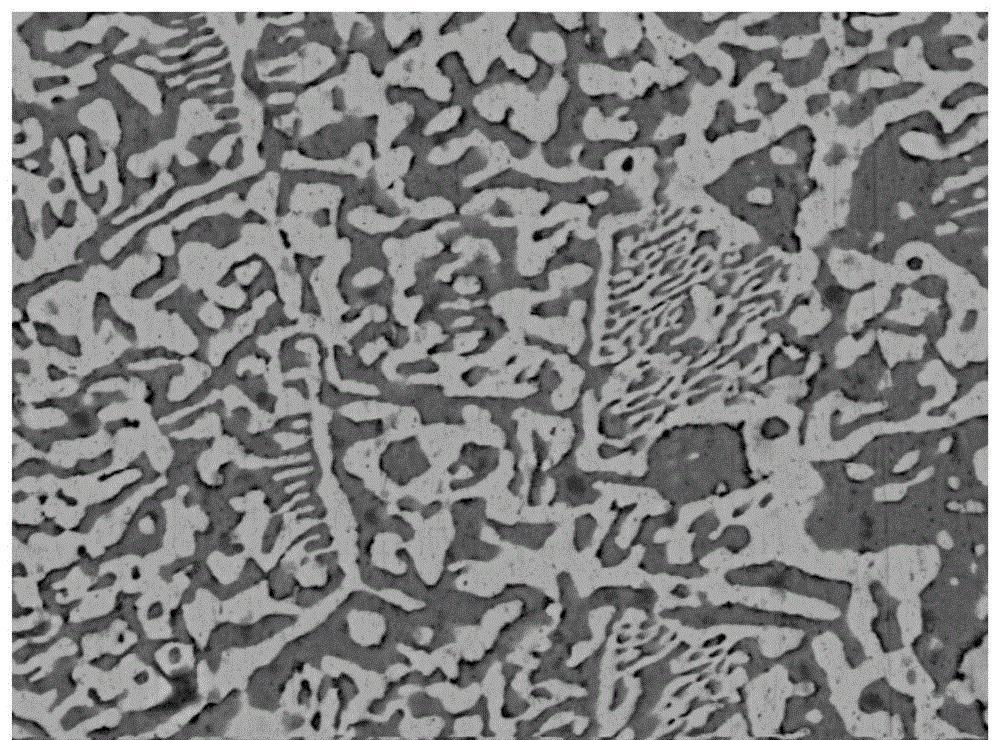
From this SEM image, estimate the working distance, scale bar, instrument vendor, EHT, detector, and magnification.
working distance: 11.8 mm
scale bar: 20000 nm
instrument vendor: FEI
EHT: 20 kV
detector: vCD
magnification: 5 K X